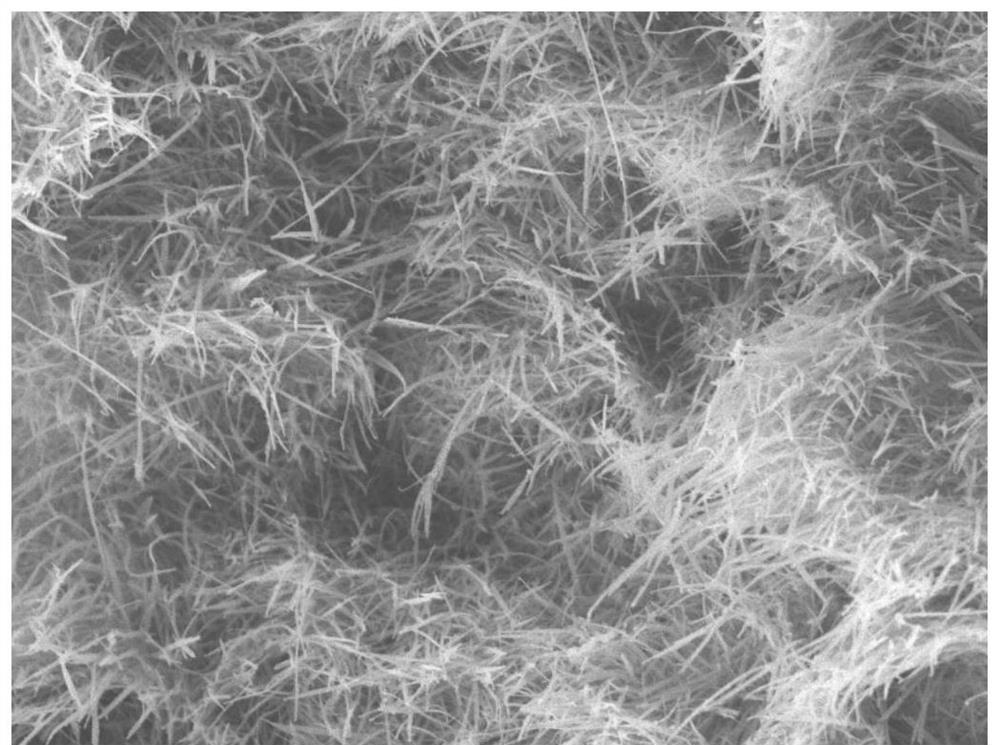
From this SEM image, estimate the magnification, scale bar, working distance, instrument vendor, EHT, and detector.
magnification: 10 K X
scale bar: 1000 nm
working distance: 7.9 mm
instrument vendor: JEOL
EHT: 5 kV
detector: LEI